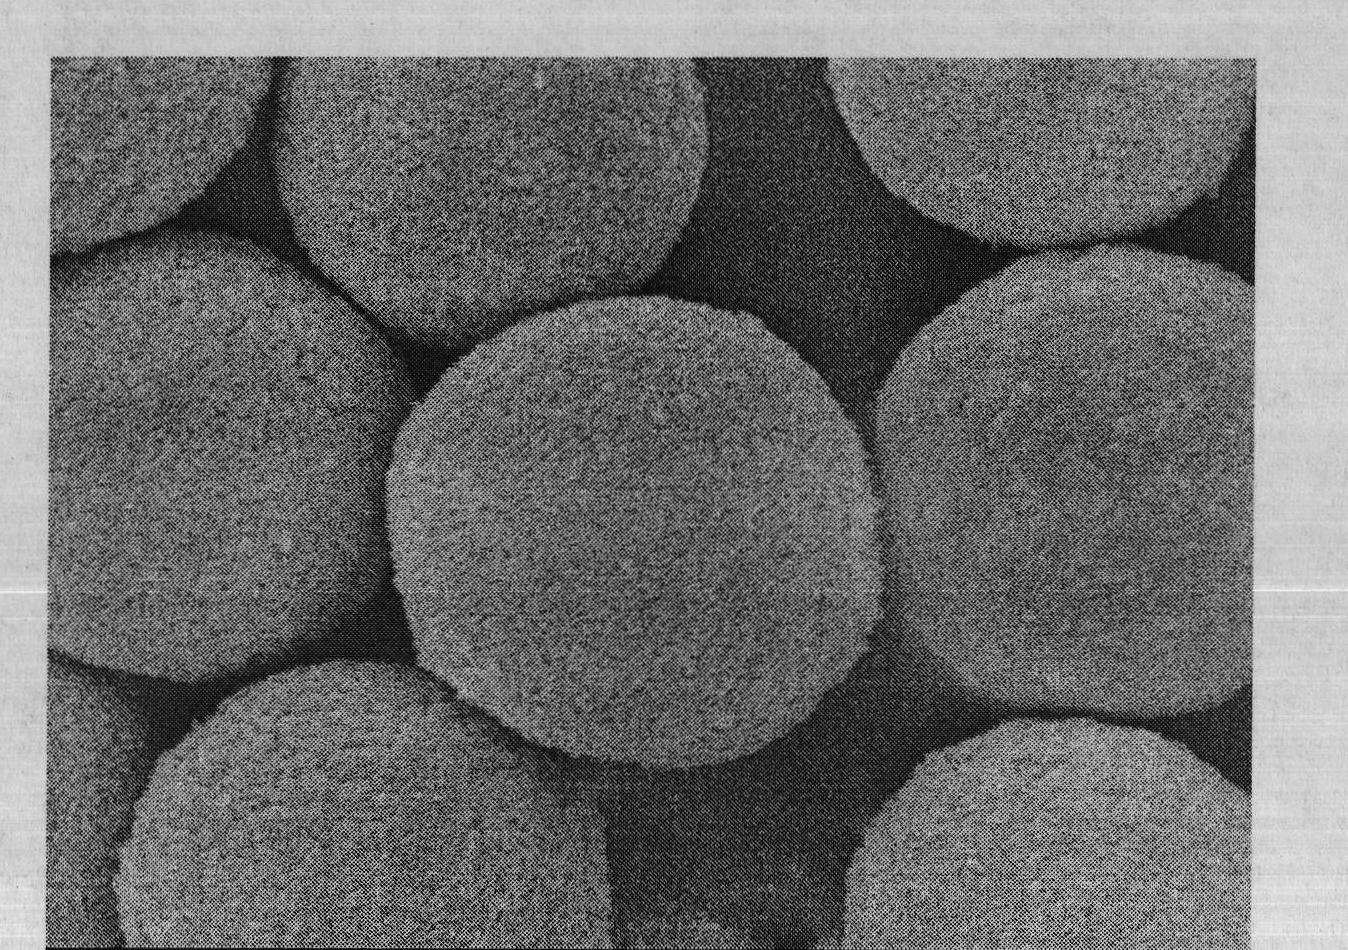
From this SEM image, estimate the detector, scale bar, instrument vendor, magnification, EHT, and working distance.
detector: SEI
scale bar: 1000 nm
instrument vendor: JEOL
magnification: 10 K X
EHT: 8 kV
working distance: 7.8 mm